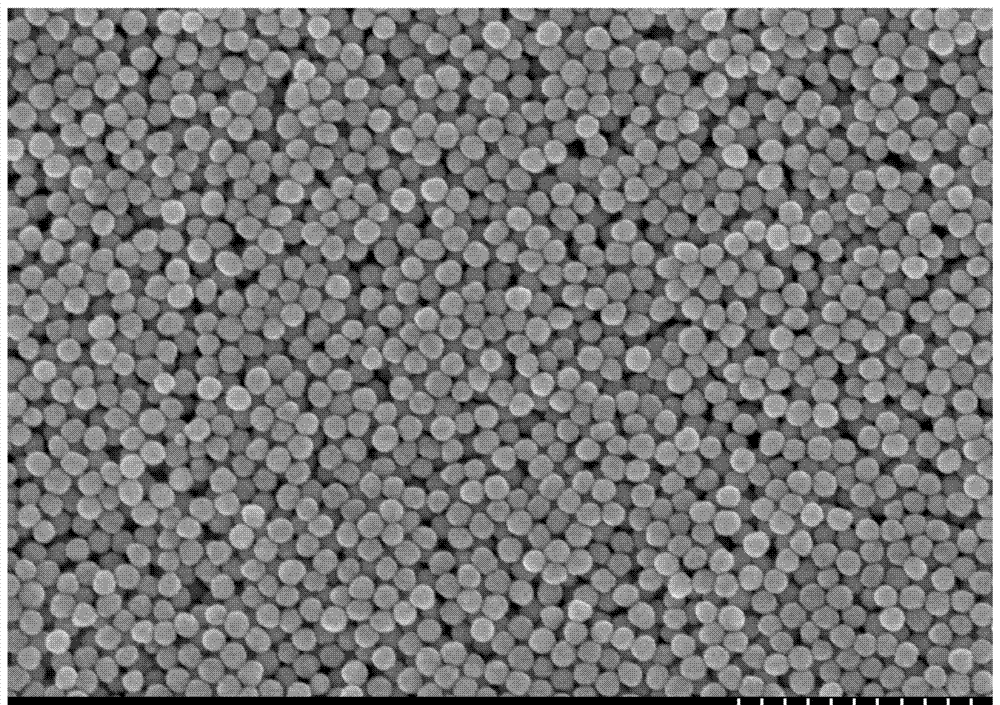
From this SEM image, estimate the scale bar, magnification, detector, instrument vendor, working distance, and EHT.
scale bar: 1000 nm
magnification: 30.1 K X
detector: SE(M)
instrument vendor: Hitachi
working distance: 8.8 mm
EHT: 15 kV